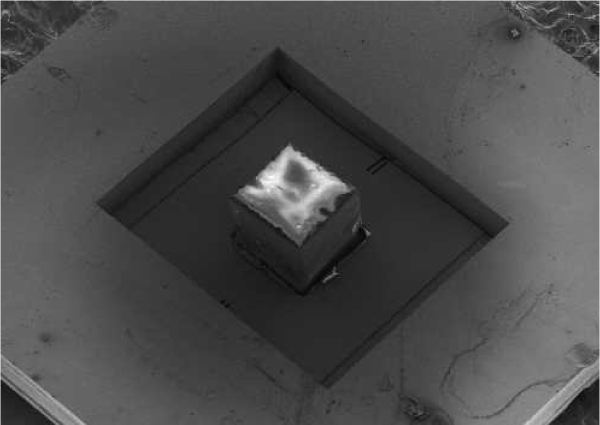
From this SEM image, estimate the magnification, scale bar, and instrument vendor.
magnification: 0.02 K X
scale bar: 1000000 nm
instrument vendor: JEOL